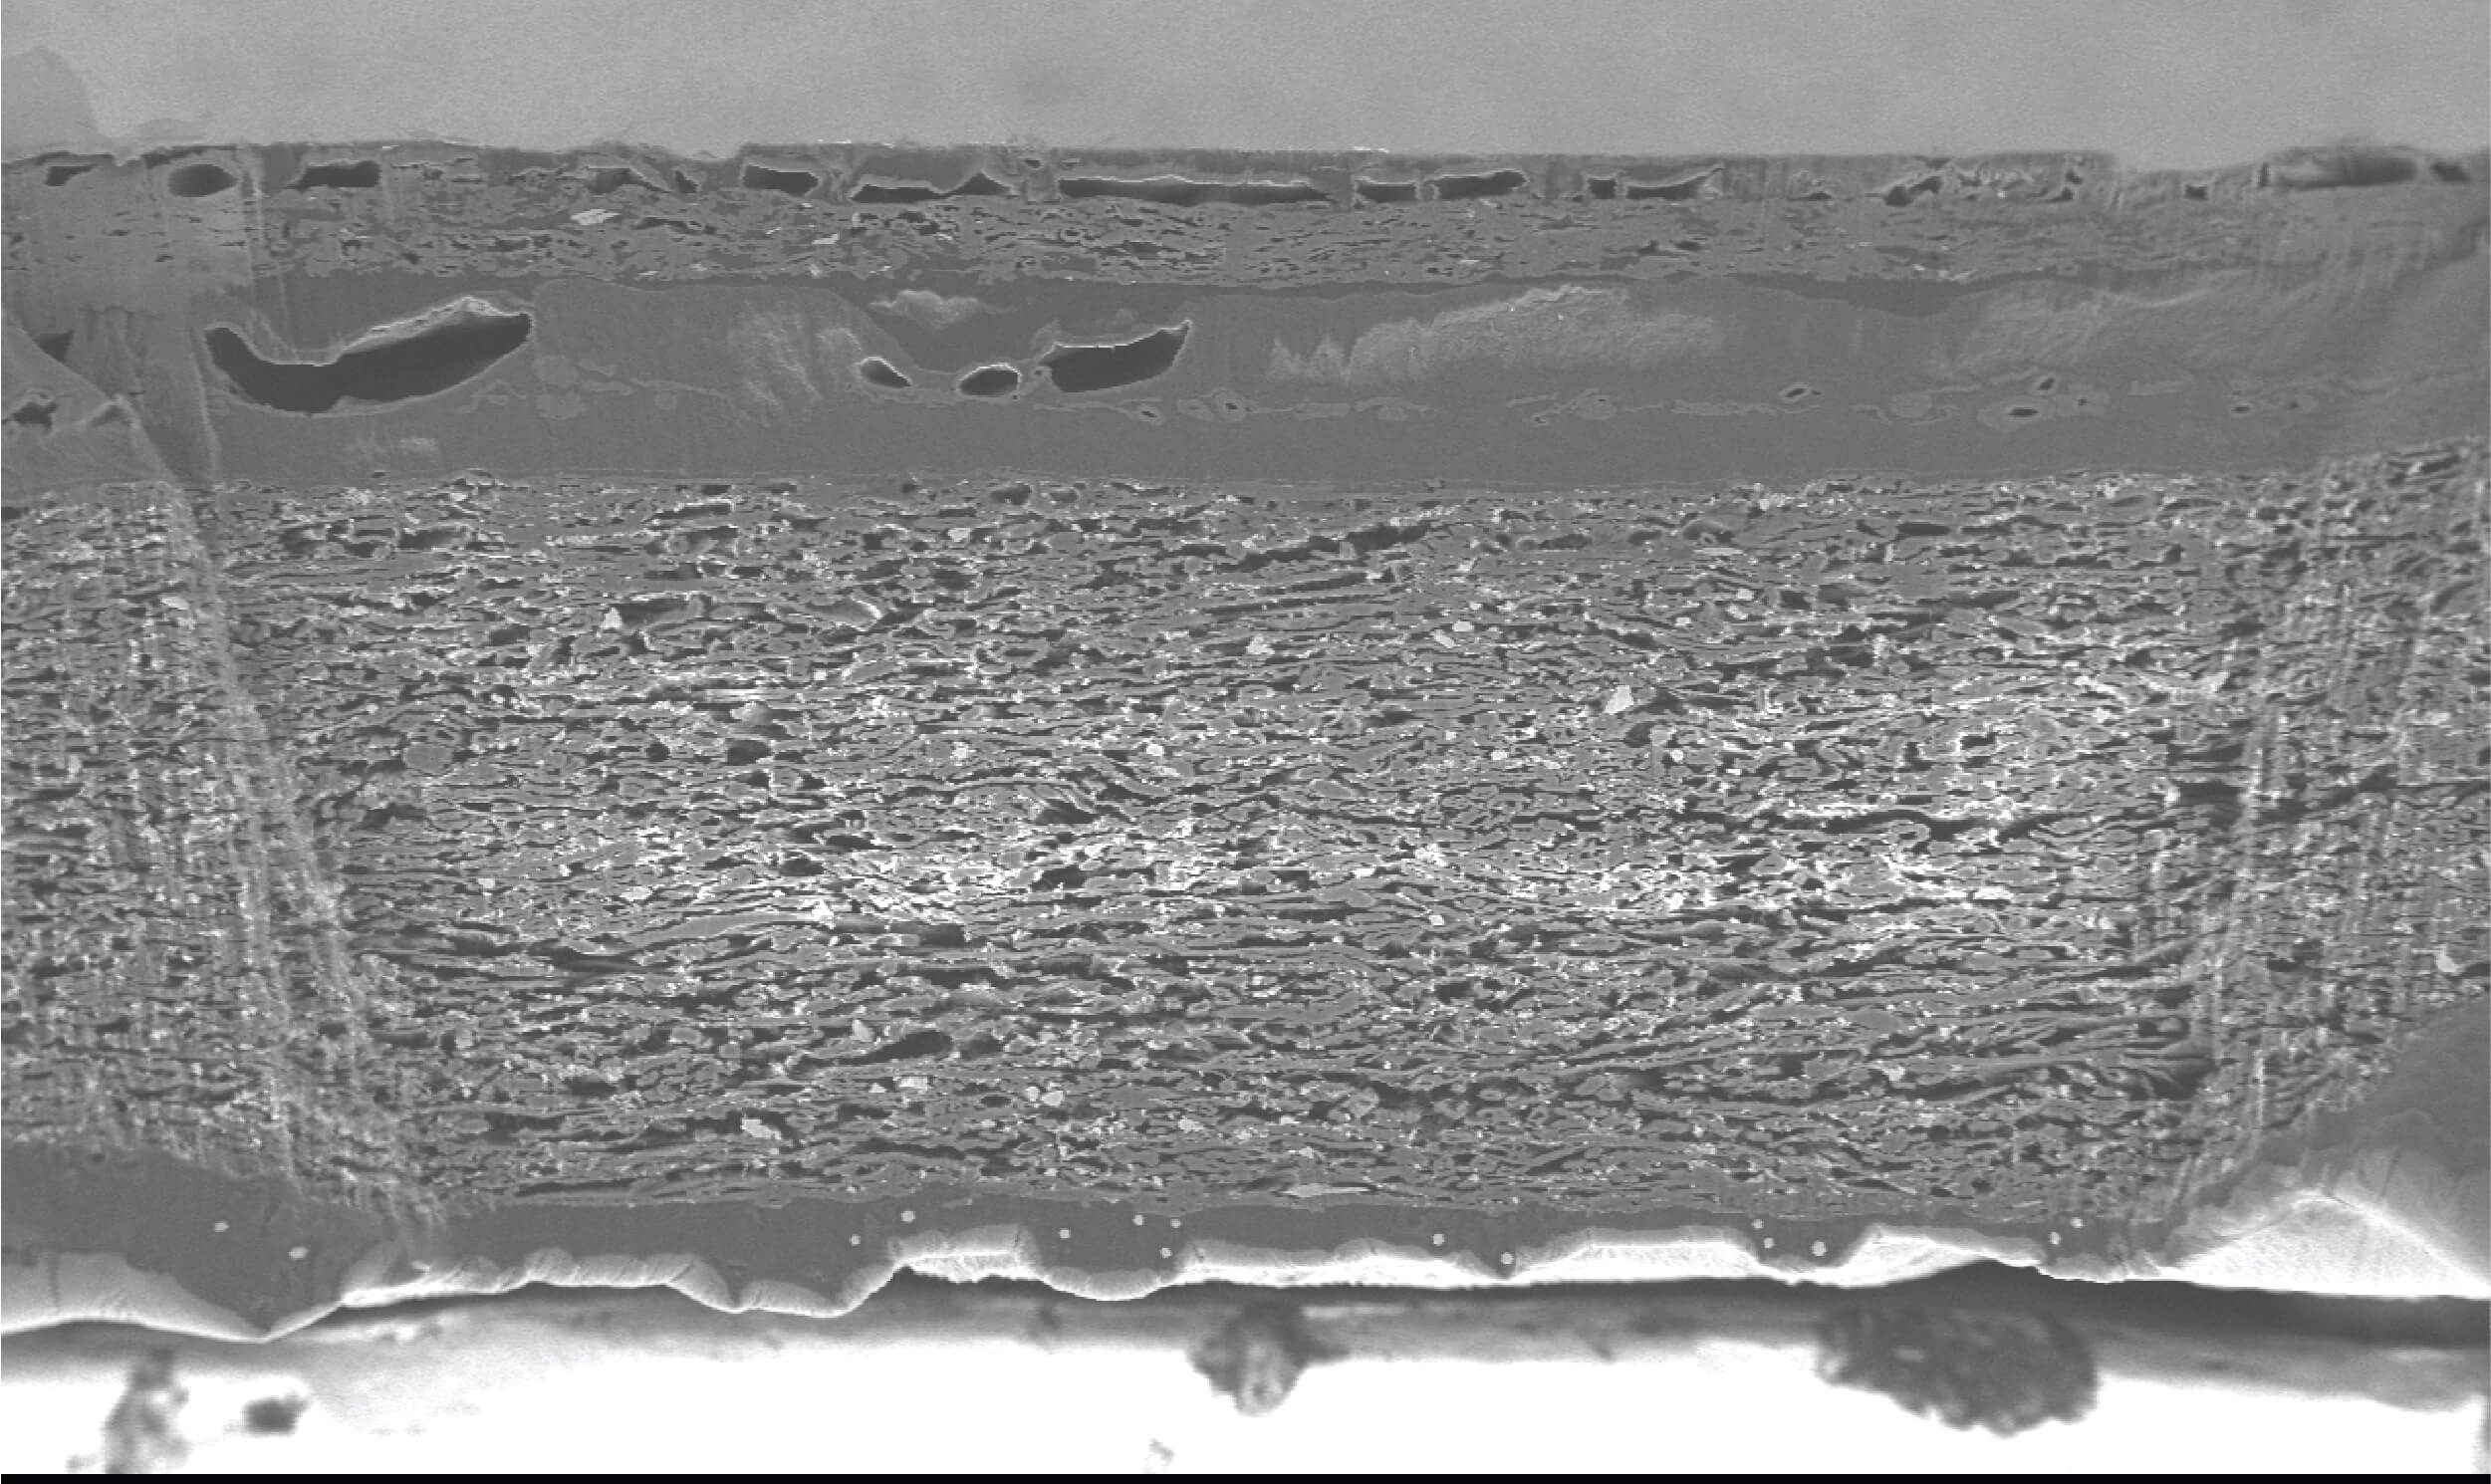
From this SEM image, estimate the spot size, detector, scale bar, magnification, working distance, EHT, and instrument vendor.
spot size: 8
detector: BSE-COMPO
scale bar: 500000 nm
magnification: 0.13 K X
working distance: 8.3 mm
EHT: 20 kV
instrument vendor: JEOL